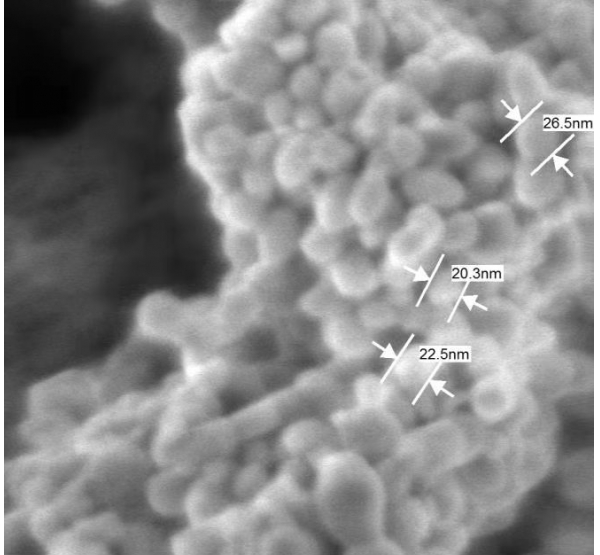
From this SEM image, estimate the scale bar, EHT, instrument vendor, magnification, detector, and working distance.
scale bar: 100 nm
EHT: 1 kV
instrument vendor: JEOL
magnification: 270 K X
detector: SEI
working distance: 3.1 mm